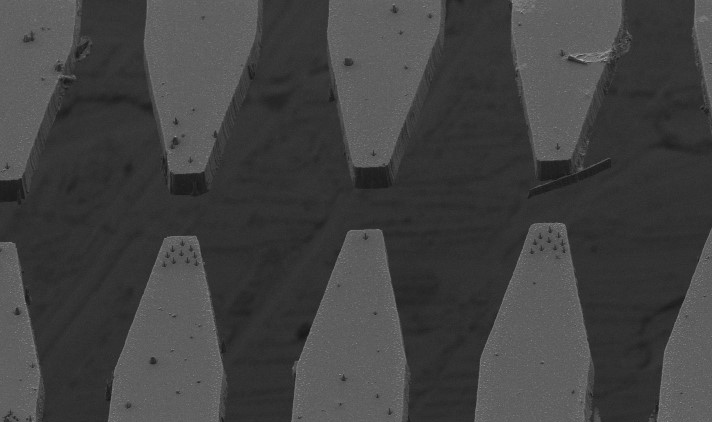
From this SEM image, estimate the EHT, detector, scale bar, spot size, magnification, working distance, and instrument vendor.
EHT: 2 kV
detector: SE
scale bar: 20000 nm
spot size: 3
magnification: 2 K X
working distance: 12.9 mm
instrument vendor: FEI